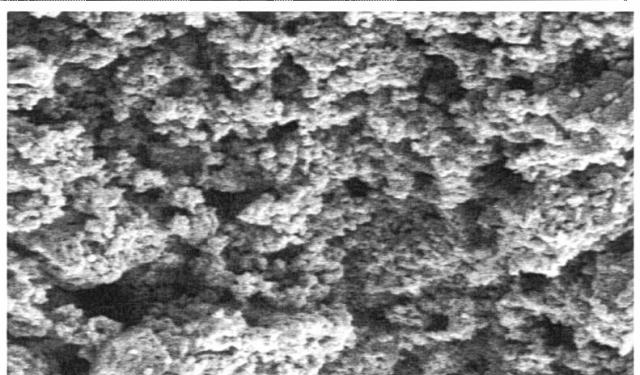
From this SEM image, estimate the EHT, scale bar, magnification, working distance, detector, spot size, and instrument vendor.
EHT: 5 kV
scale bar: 2000 nm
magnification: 20 K X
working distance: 3.8 mm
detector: SE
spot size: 3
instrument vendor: FEI